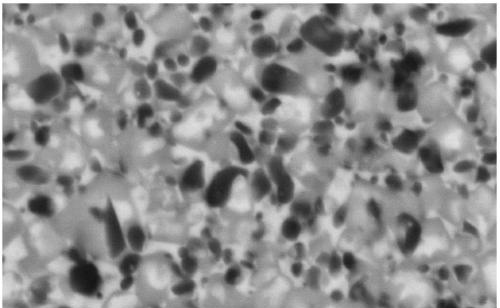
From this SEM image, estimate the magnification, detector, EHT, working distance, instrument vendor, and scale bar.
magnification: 10 K X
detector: NTS BSD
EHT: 20 kV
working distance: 9.5 mm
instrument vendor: Zeiss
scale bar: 1000 nm